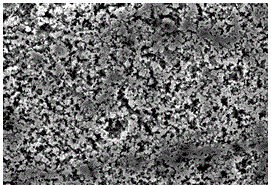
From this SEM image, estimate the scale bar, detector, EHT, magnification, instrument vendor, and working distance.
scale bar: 1000 nm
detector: InLens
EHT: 6 kV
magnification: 5 K X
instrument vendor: Zeiss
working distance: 7.5 mm